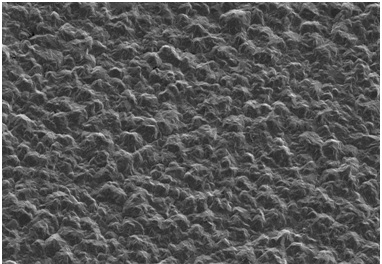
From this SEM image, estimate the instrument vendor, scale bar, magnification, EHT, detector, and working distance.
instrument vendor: Hitachi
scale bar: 50000 nm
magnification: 1 K X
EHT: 3 kV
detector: SE(UL)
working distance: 34.9 mm